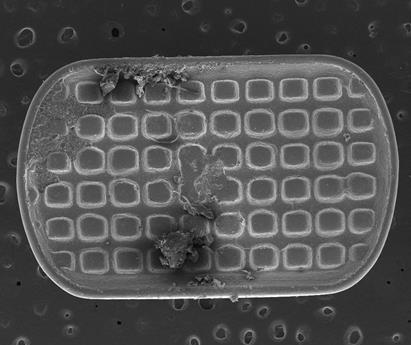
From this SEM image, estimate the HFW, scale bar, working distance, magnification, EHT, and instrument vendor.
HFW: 4260 µm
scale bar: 2000000 nm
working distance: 30.7 mm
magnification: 0.07 K X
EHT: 20 kV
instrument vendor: FEI